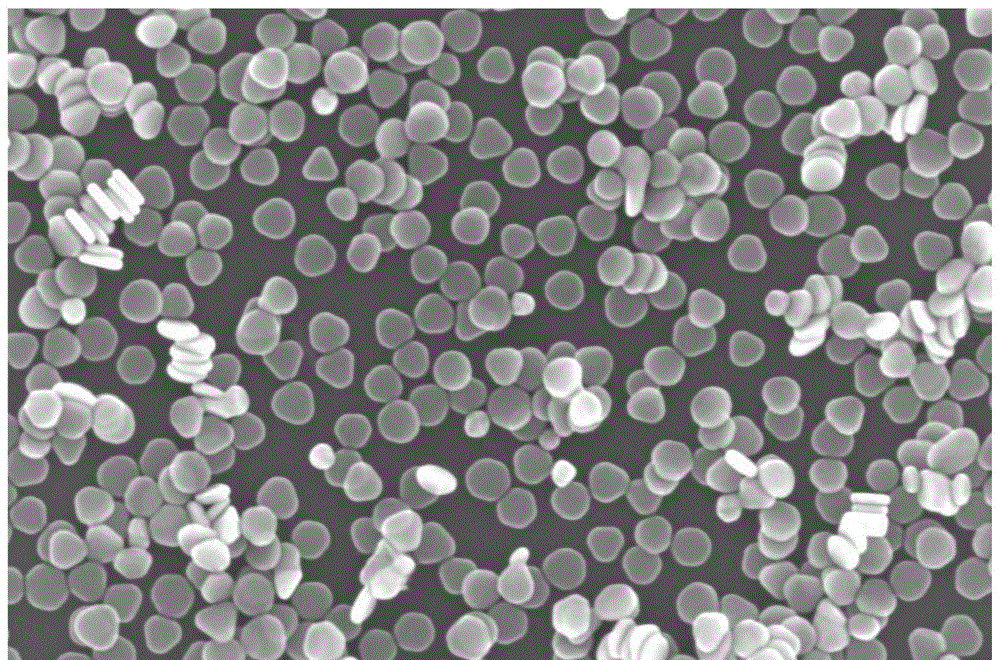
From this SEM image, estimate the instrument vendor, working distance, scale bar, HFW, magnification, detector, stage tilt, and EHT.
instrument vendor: FEI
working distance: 3.9 mm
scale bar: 500 nm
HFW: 1.73 µm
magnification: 240 K X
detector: TLD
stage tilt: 0°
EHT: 10 kV